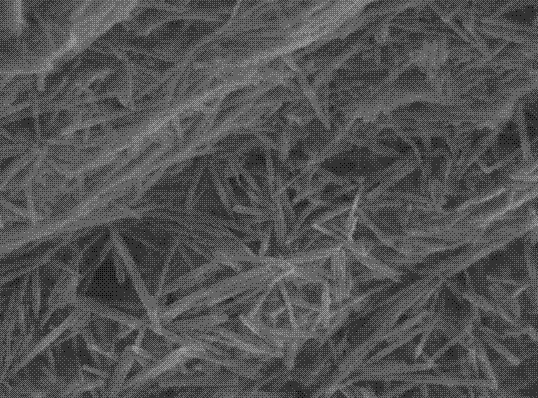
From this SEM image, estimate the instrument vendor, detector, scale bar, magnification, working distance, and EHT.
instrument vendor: JEOL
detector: SEI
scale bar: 1000 nm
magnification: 20 K X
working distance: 9.9 mm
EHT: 5 kV